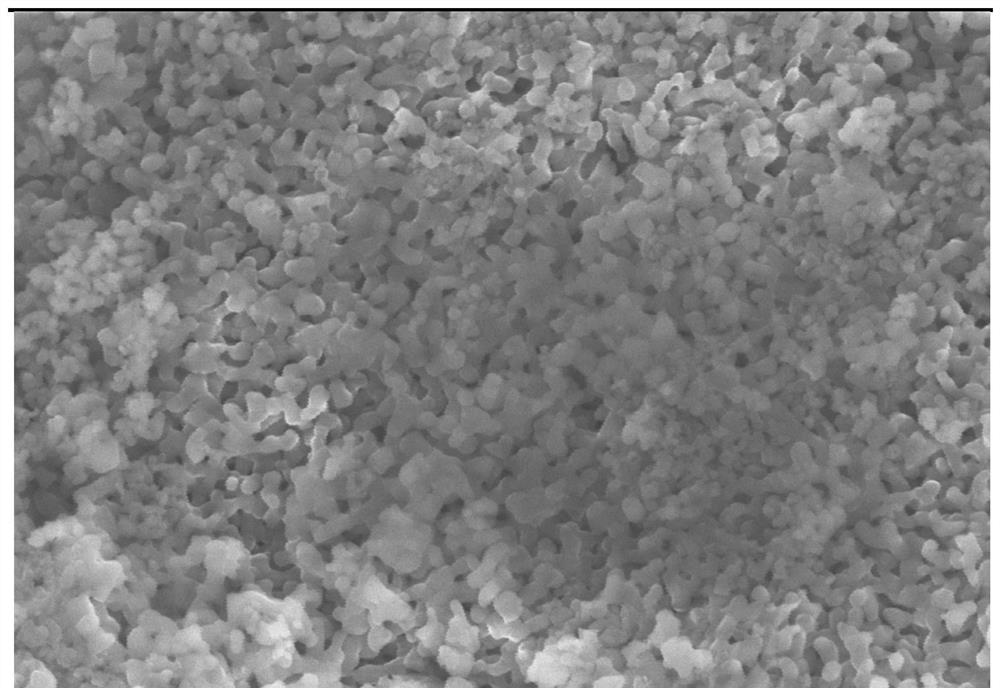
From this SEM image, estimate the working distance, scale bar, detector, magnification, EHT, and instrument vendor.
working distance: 9.2 mm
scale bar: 200 nm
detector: InLens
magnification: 20 K X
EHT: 10 kV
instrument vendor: Zeiss